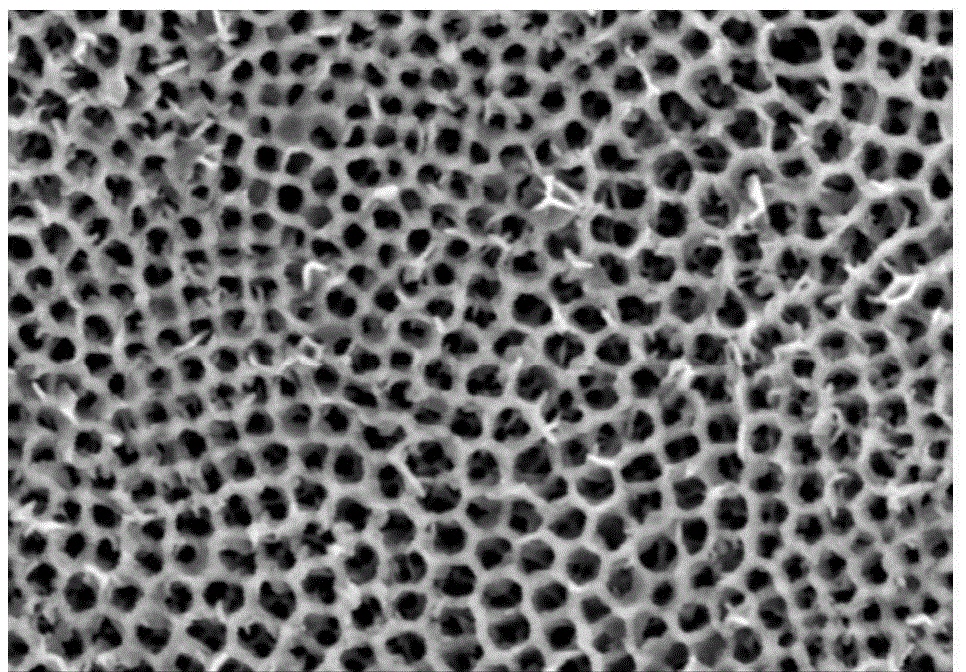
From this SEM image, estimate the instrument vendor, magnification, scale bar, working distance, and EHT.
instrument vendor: Hitachi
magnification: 40 K X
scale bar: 1000 nm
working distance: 15.7 mm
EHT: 3 kV